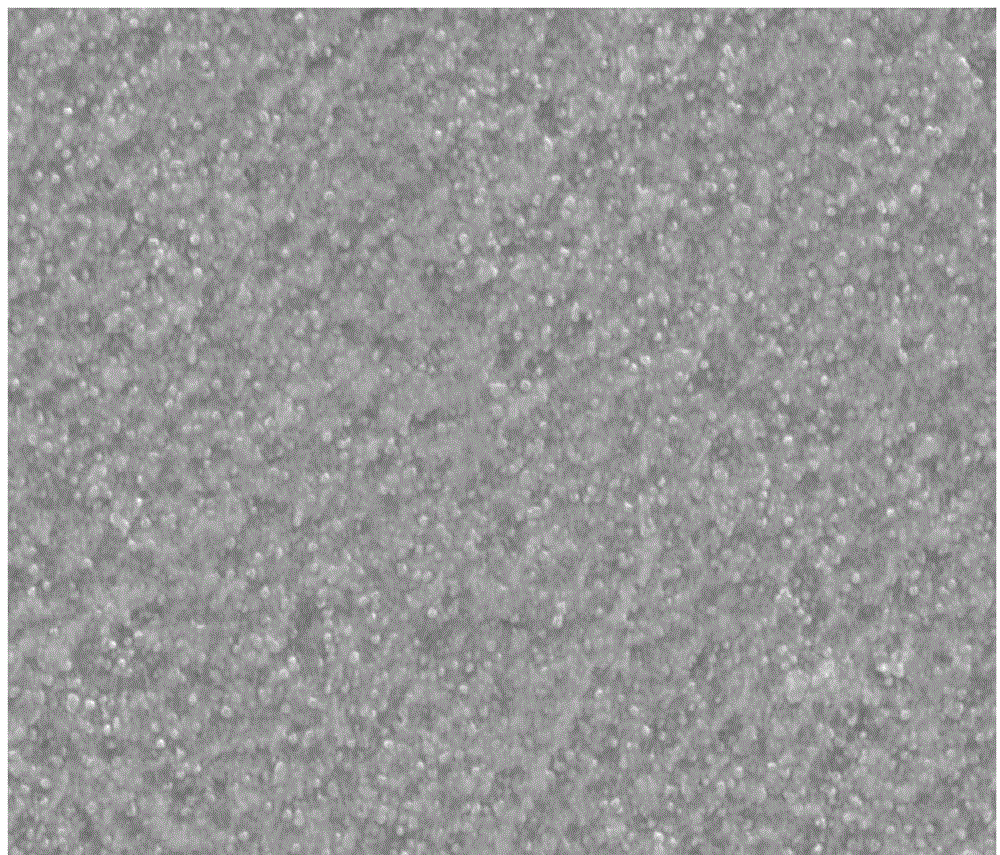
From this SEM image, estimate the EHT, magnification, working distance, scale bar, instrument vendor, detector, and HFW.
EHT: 20 kV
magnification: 10 K X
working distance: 10.2 mm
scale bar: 5000 nm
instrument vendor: FEI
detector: ETD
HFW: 12.7 µm